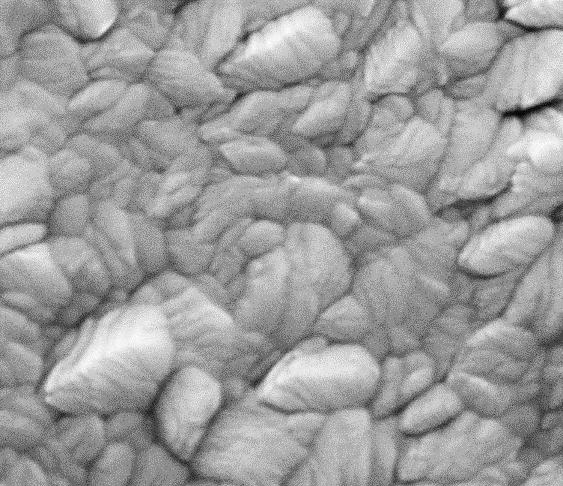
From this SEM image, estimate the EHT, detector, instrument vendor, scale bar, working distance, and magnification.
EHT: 15 kV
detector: ETD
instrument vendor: FEI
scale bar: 500 nm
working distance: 8.2 mm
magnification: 200 K X